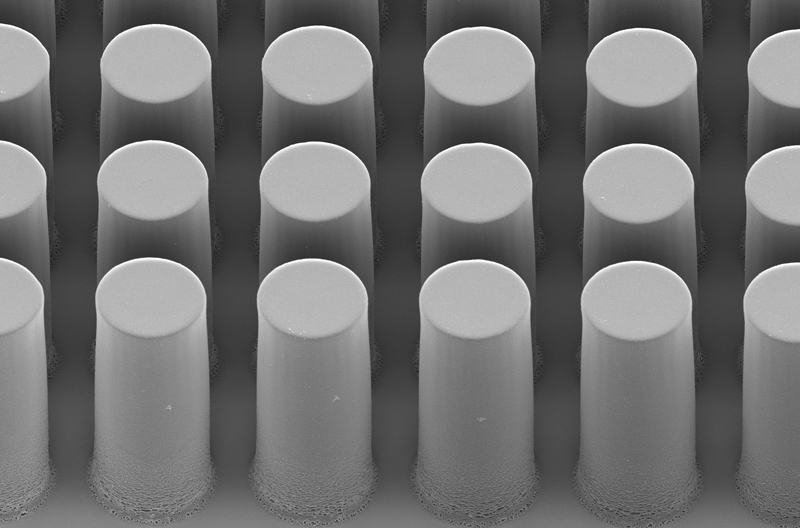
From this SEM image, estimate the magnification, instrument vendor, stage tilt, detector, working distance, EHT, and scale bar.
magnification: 0.791 K X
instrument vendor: Zeiss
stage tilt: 45°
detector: InLens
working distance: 9.5 mm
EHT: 3 kV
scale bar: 20000 nm